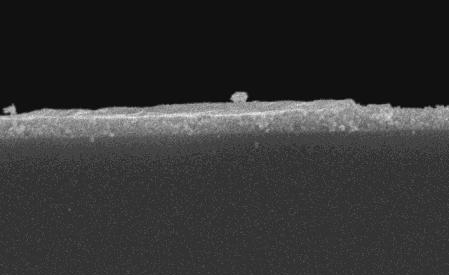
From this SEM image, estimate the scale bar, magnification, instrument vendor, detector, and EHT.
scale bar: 5000 nm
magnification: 5 K X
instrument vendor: JEOL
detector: SEI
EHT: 20 kV